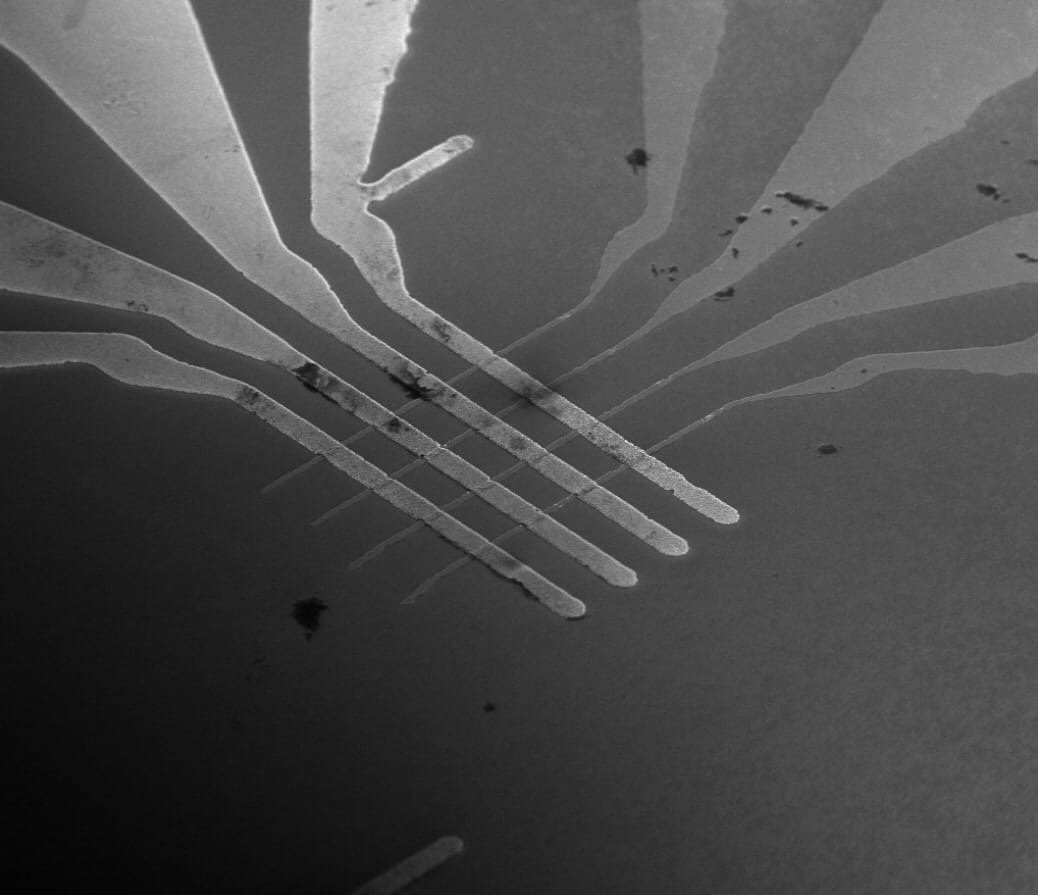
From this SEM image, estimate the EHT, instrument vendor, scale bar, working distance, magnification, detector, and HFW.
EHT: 10 kV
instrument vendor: FEI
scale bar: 30000 nm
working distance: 5.1 mm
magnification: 2.5 K X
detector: TLD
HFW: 102 µm